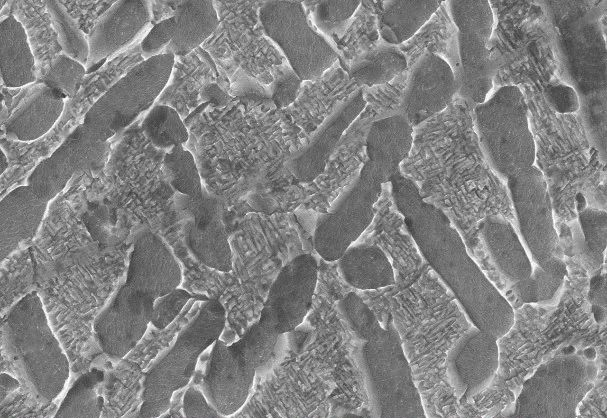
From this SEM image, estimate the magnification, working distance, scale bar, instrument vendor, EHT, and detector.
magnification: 10 K X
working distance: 8.4 mm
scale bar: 5000 nm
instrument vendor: other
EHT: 25 kV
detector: SE